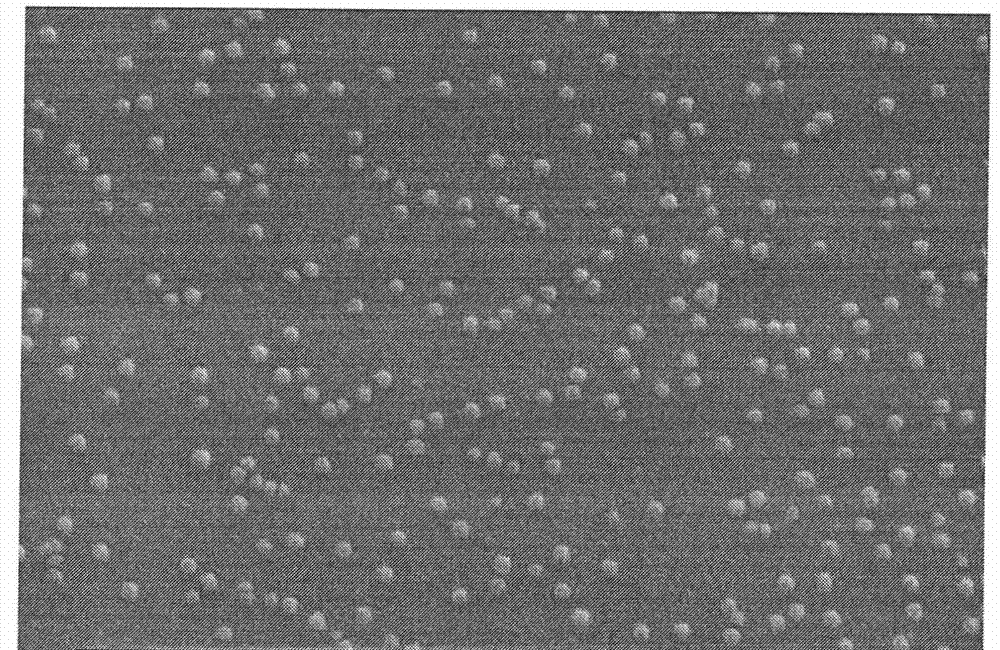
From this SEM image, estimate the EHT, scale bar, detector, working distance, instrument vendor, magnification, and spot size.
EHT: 25 kV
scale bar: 500 nm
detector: SE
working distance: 5.6 mm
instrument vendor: FEI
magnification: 100 K X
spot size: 4